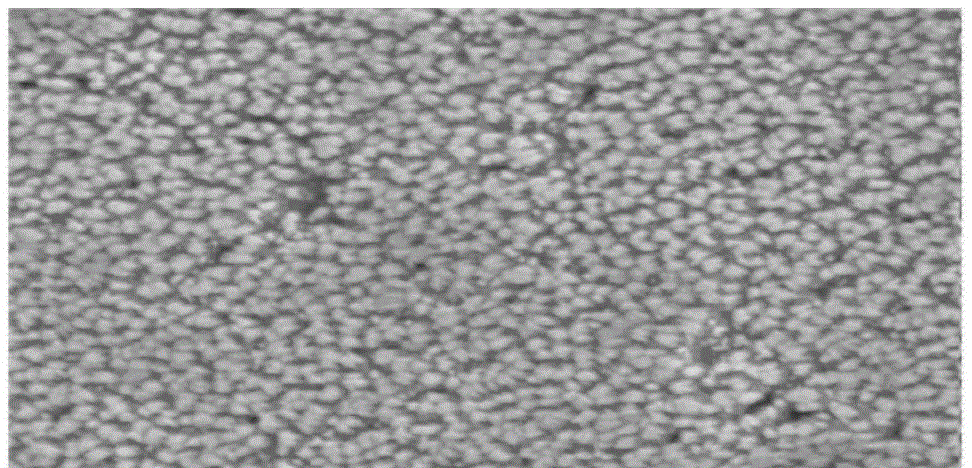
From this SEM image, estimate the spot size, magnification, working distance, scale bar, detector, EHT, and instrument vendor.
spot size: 3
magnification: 100 K X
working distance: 5.7 mm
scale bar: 500 nm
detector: SE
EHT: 5 kV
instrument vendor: FEI